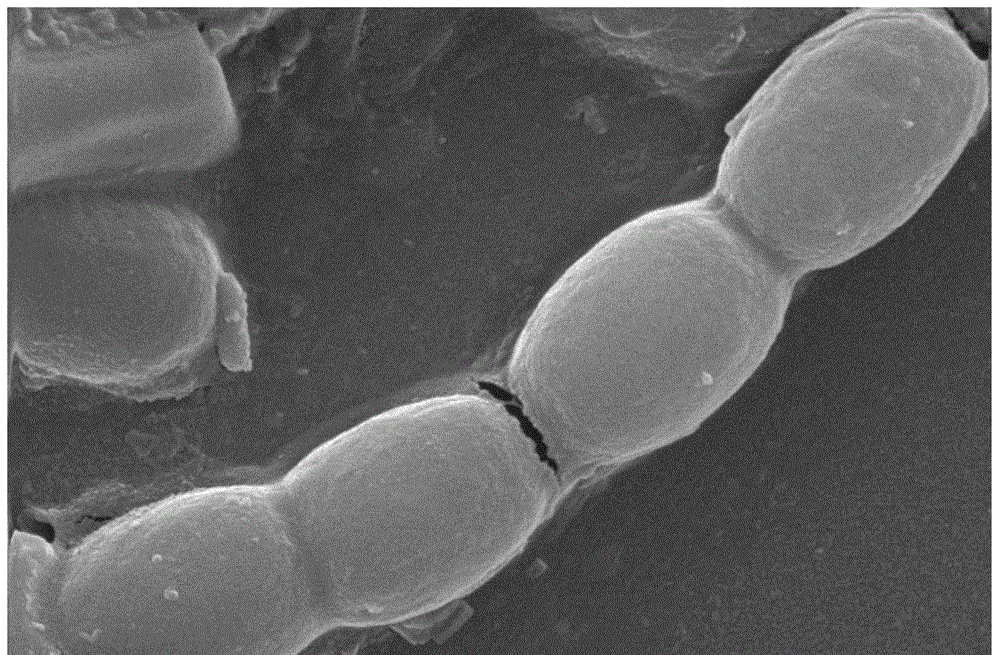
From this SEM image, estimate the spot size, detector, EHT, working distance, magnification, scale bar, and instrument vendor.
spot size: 3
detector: SE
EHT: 20 kV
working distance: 5.6 mm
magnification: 10 K X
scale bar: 2000 nm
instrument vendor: FEI